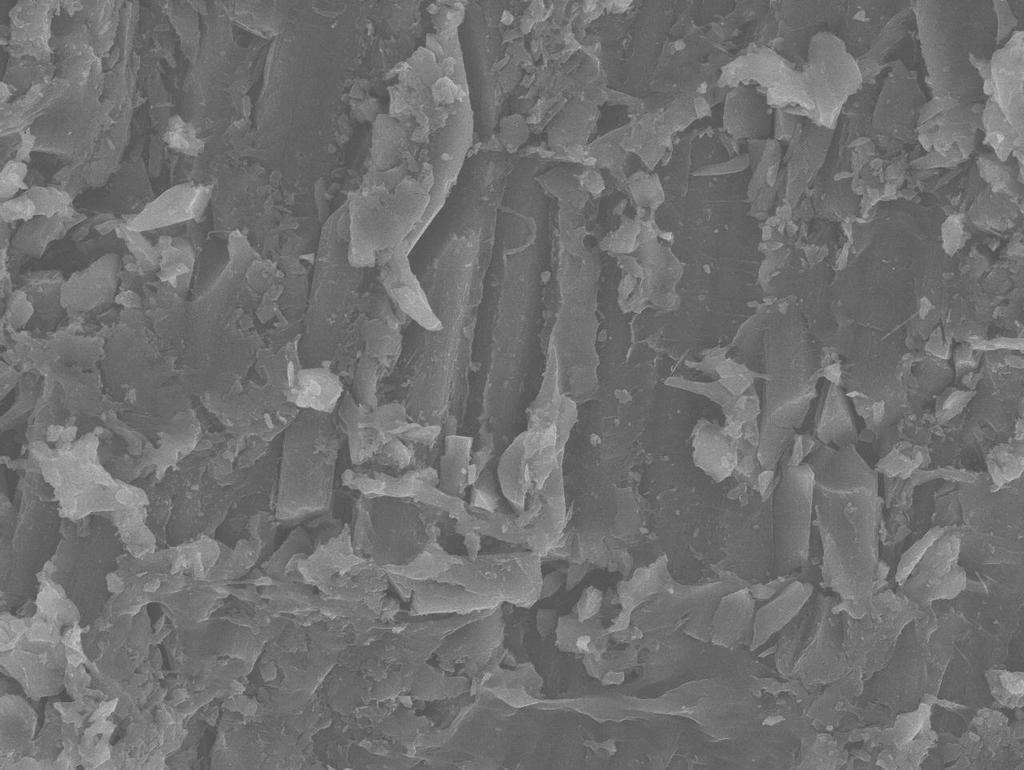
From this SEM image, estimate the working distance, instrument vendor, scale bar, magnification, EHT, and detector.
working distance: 39.4 mm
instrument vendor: JEOL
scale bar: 10000 nm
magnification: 1 K X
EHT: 20 kV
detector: SEI